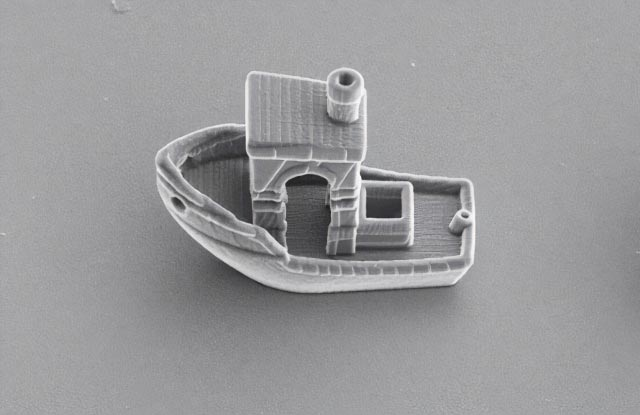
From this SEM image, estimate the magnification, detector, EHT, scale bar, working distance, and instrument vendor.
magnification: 3.5 K X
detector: ETD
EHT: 5 kV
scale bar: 20000 nm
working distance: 12.9 mm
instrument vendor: Thermo